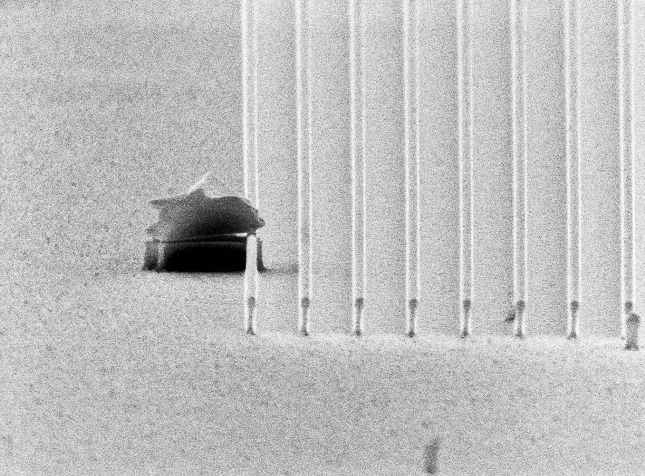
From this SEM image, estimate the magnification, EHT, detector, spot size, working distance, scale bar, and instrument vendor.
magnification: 13.234 K X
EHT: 10 kV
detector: SE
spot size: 3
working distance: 21.5 mm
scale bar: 2000 nm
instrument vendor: FEI